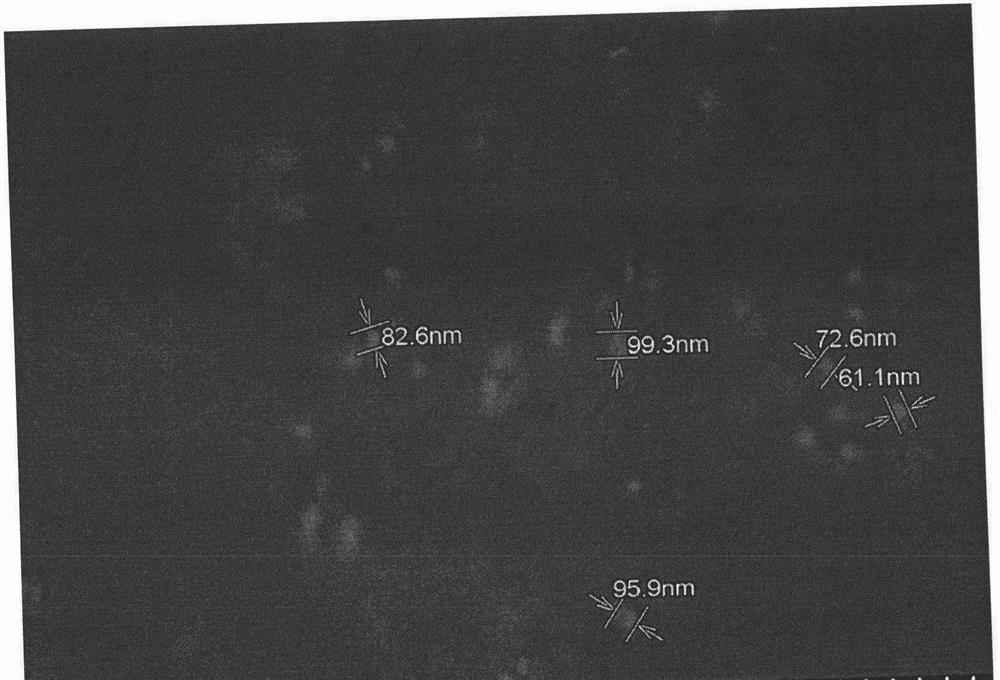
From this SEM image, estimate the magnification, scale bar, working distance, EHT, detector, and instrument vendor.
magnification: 35 K X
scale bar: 1000 nm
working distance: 7.3 mm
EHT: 5 kV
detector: SE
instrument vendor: Hitachi